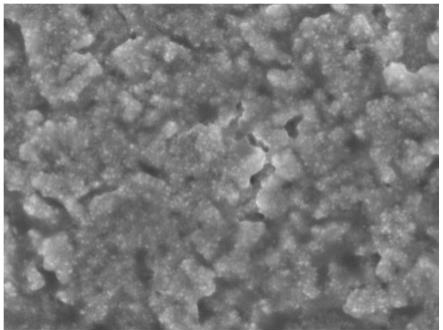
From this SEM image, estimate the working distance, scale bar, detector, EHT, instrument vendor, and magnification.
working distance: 7.3 mm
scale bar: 100 nm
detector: SEI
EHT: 5 kV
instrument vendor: JEOL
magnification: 50 K X